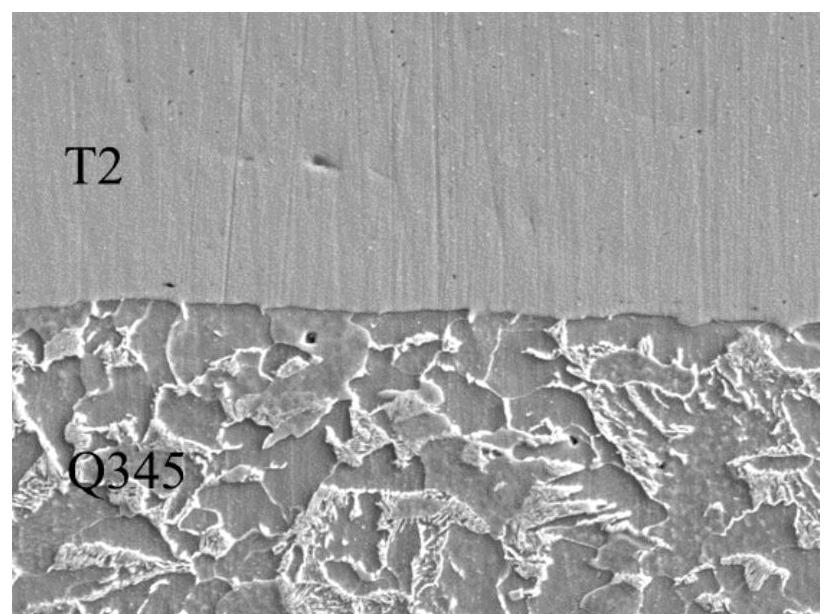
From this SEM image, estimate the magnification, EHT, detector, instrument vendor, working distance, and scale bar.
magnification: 2 K X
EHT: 20 kV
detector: SEI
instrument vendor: JEOL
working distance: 10.9 mm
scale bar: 10000 nm